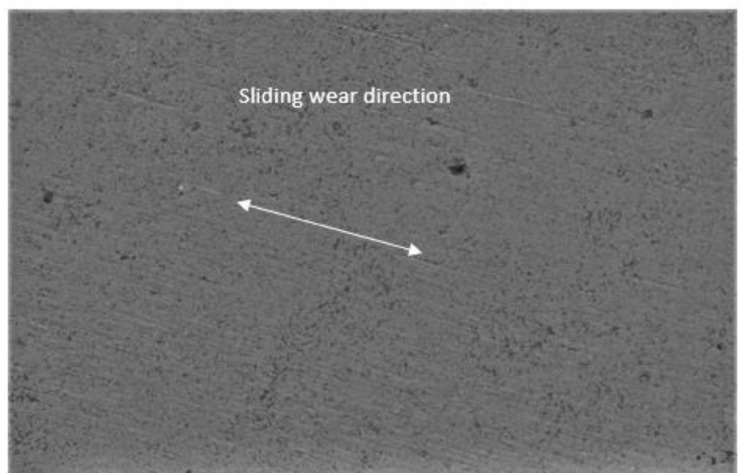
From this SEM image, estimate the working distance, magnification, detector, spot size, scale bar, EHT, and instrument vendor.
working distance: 13.6 mm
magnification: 1 K X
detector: SE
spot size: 2.5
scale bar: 20000 nm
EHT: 10 kV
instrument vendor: FEI